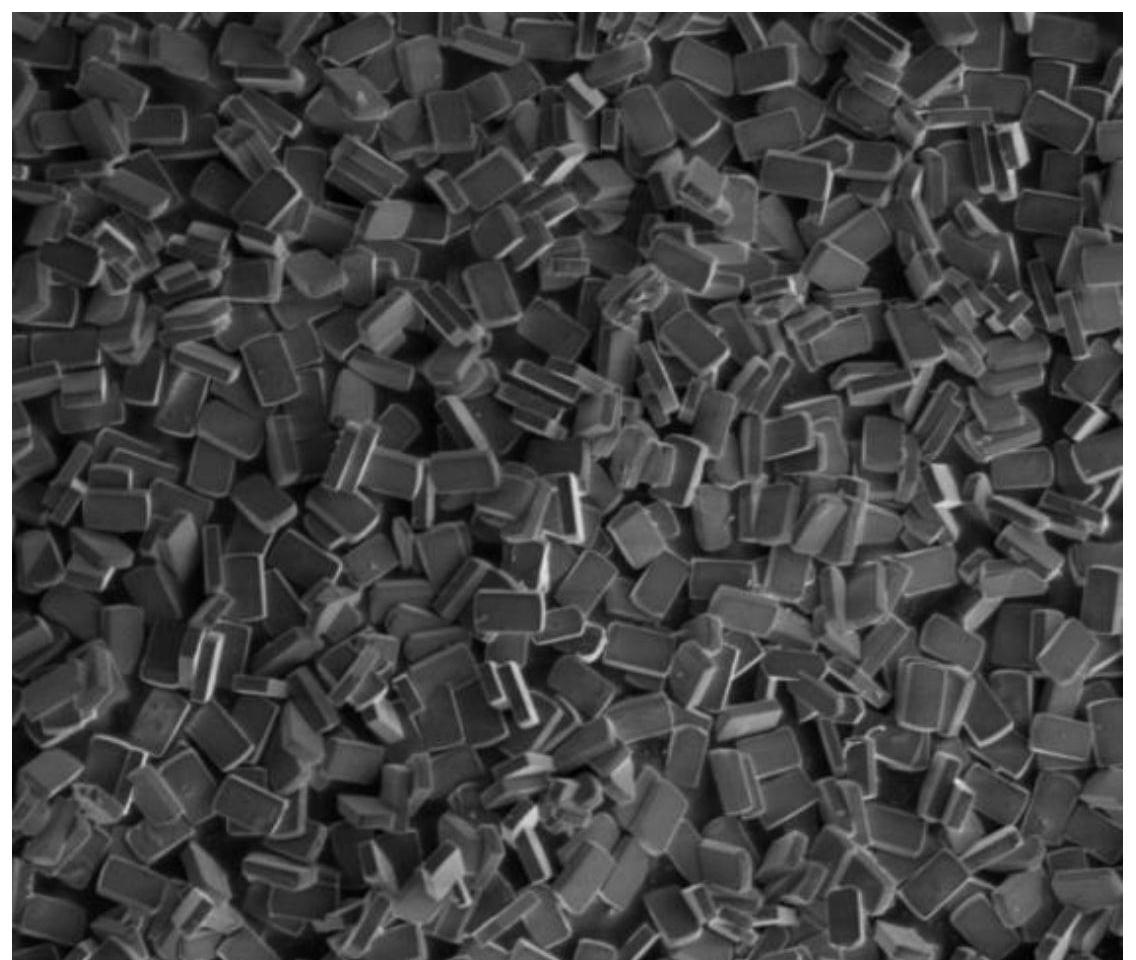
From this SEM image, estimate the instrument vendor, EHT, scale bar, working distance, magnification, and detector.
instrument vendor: FEI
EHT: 3 kV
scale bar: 10000 nm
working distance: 5.1 mm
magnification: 5 K X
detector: SE